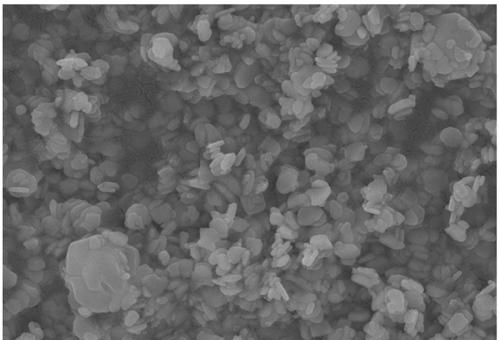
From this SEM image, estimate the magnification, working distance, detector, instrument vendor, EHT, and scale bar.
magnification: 29.38 K X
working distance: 11.2 mm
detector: SE2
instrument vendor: Zeiss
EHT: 15 kV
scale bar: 200 nm